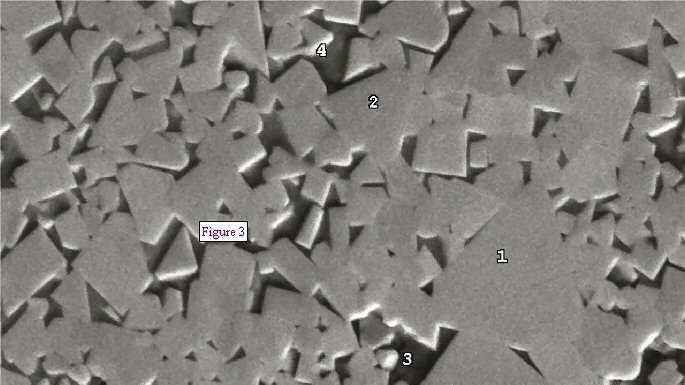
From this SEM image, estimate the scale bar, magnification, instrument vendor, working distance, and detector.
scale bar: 10000 nm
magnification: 3.5 K X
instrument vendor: JEOL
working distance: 14.9 mm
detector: BSE1